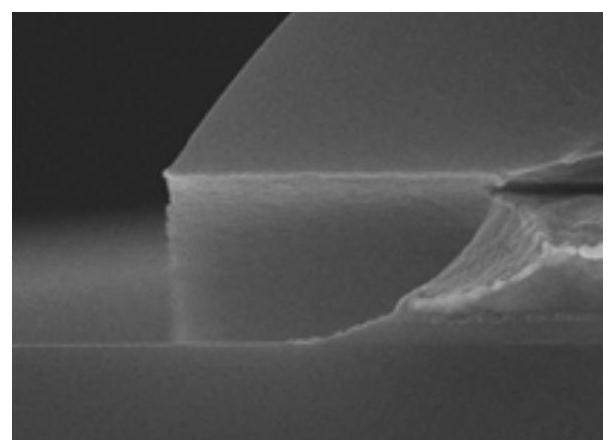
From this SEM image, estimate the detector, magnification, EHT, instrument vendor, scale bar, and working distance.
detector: SE(U)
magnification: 50 K X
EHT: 10 kV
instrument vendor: Hitachi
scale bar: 1000 nm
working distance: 10.8 mm